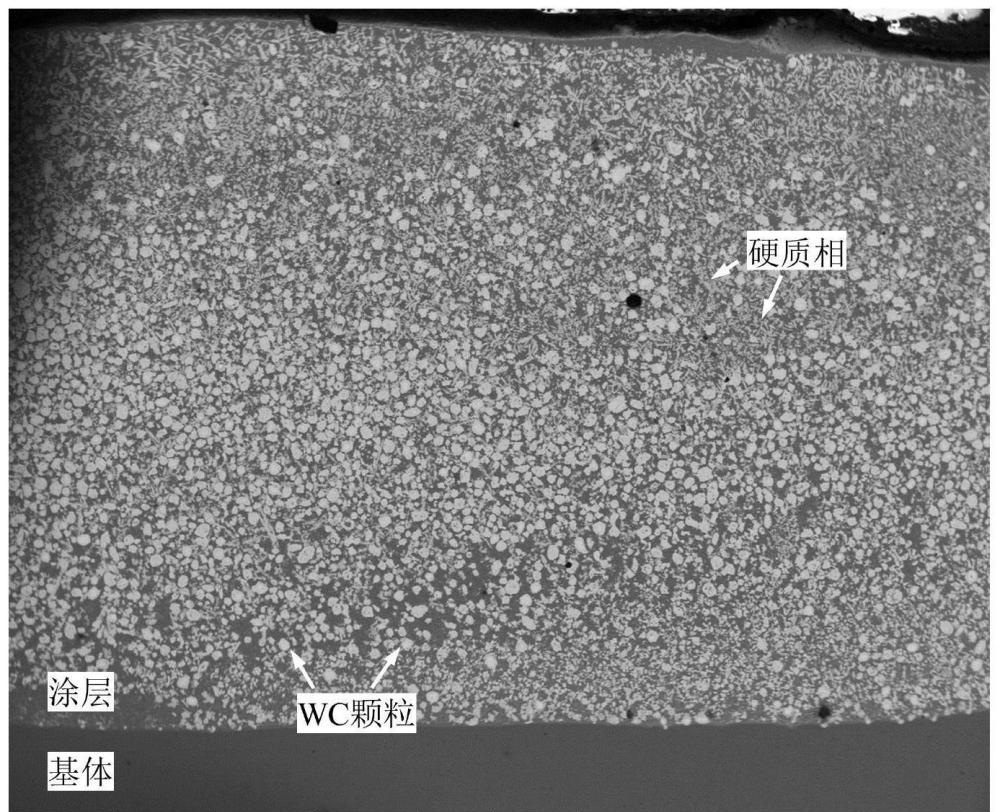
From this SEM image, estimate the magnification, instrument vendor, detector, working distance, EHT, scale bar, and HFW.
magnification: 0.08 K X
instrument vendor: FEI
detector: BSED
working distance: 9.5 mm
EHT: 20 kV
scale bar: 1000000 nm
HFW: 3730 µm